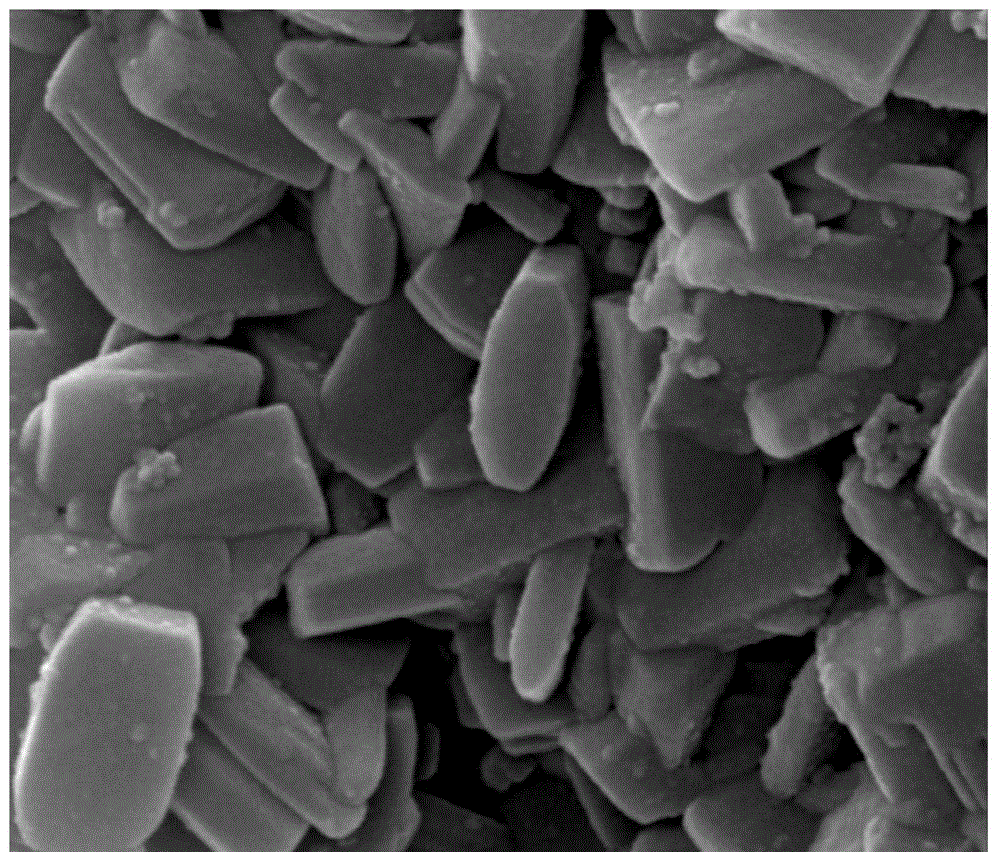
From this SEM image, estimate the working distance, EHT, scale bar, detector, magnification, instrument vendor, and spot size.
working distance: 10 mm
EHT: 20 kV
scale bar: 500 nm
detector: ETD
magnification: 100 K X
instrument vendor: FEI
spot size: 3